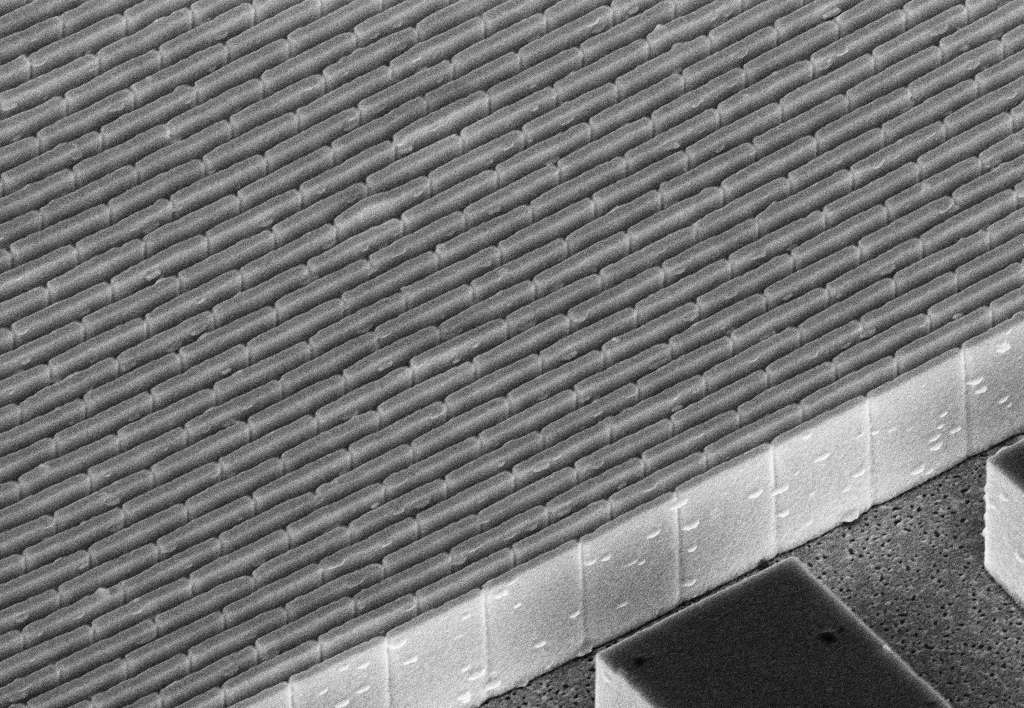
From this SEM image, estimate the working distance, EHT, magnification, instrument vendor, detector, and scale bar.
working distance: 5.6 mm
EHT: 10 kV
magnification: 13.89 K X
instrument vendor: Zeiss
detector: InLens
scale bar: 200 nm